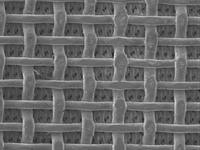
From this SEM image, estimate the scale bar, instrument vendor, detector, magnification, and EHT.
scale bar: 100000 nm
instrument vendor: JEOL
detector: LEI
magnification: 0.05 K X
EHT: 5 kV